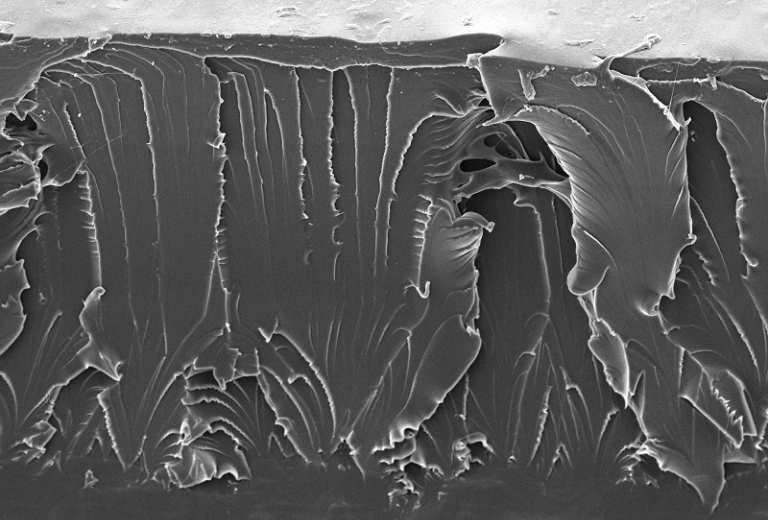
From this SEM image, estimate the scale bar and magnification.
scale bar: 200000 nm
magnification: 0.15 K X